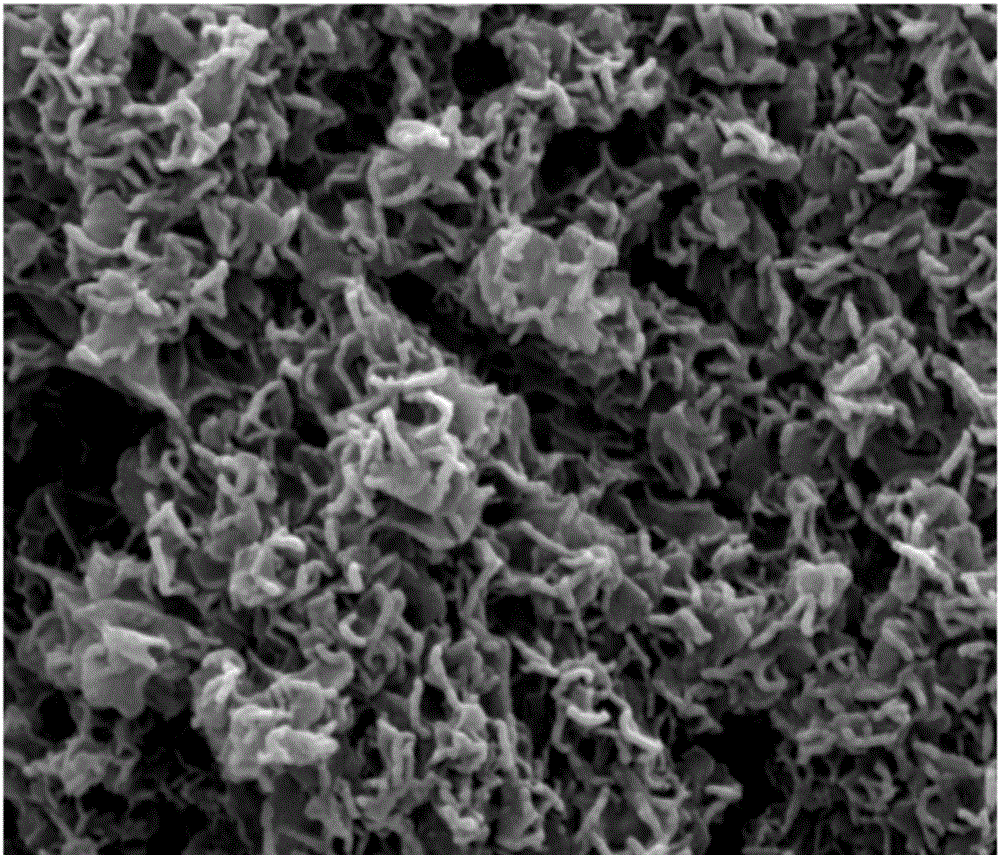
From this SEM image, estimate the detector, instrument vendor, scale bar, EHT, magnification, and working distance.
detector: SE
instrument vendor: FEI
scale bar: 1000 nm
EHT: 20 kV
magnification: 80 K X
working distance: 12.1 mm